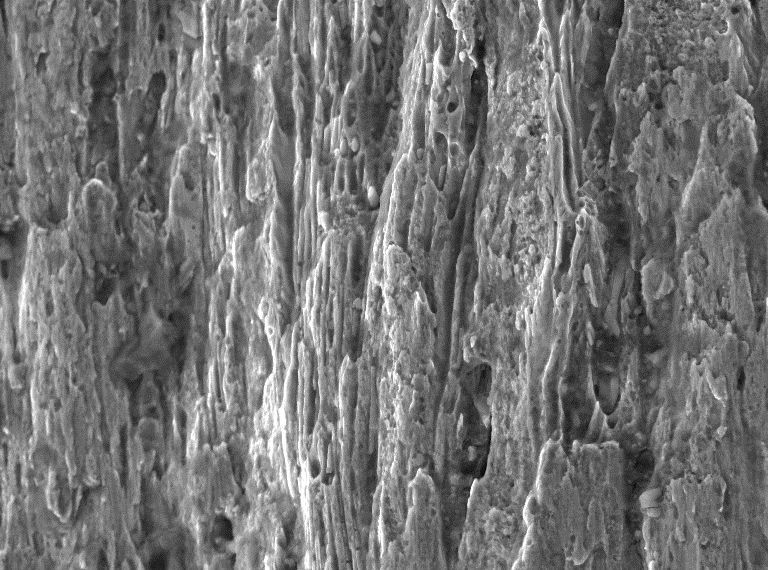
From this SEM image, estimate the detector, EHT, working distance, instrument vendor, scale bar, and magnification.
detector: SE Detector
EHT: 20 kV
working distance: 13.82 mm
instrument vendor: Tescan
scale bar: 20000 nm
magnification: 2 K X